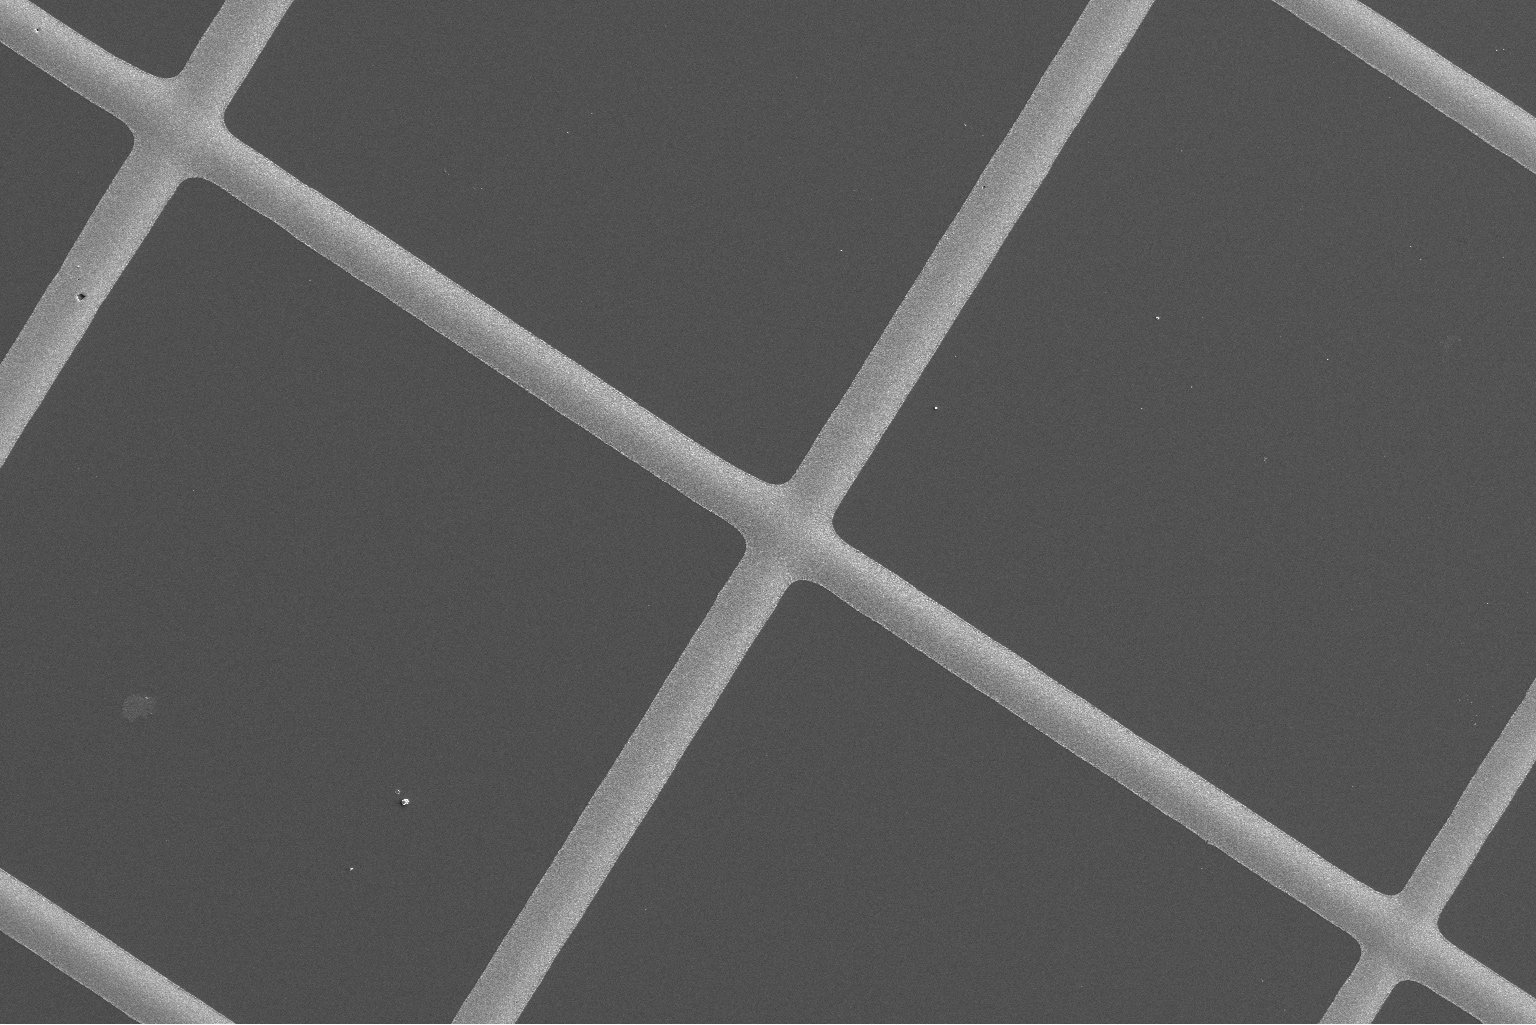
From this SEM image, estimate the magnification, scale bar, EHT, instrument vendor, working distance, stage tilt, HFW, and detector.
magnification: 1 K X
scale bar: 40000 nm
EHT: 2 kV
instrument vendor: FEI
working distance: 4 mm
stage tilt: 0°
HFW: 207 µm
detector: ETD